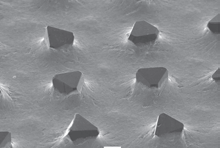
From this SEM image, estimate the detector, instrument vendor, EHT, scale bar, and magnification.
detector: SEI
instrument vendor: JEOL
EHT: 20 kV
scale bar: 100000 nm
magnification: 0.1 K X